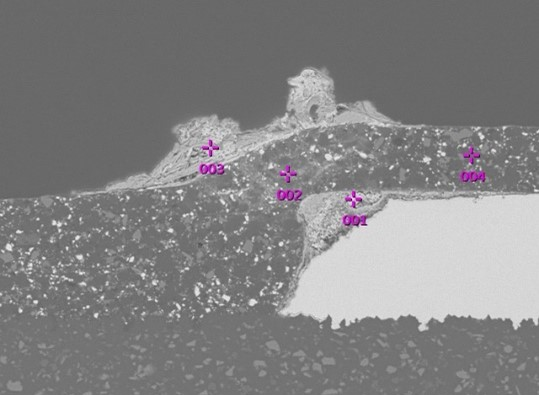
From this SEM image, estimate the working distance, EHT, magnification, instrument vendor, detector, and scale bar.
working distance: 10 mm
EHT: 15 kV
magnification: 0.5 K X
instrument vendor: JEOL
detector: BEC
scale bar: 50000 nm